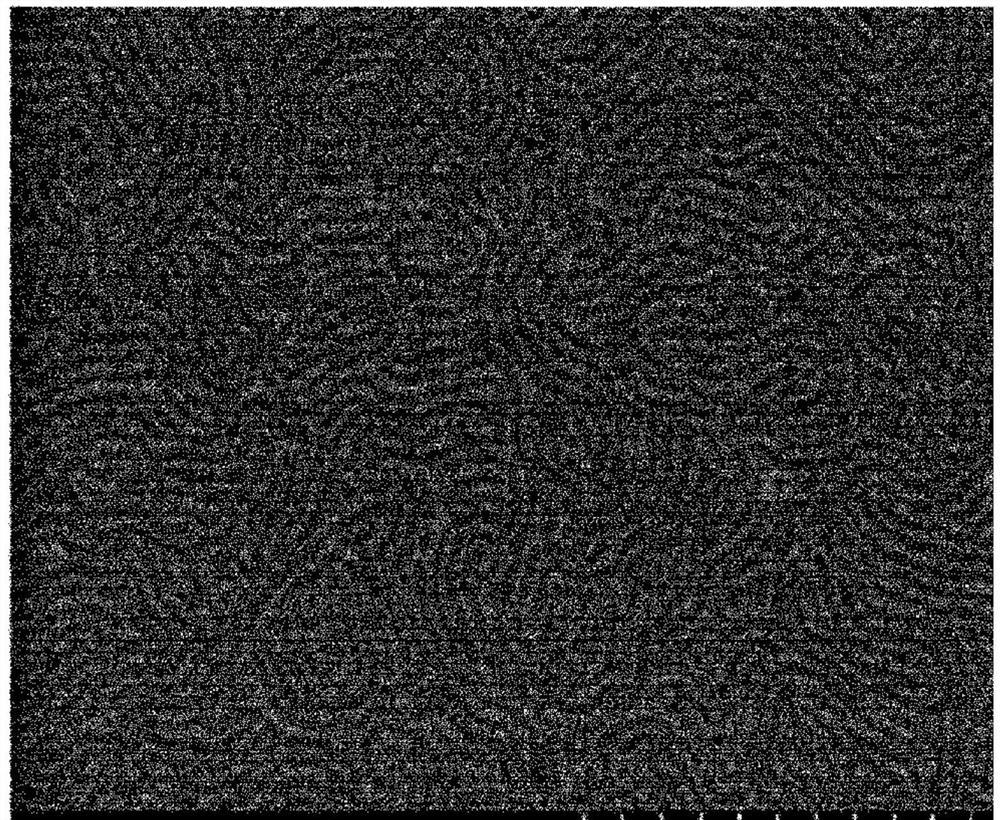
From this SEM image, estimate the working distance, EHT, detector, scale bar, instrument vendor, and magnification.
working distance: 5 mm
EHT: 2 kV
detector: SE(M)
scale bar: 500 nm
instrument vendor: Hitachi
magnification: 100 K X